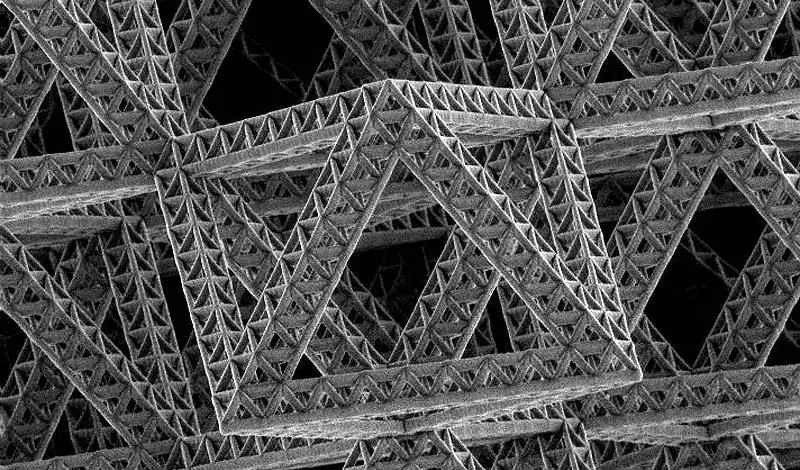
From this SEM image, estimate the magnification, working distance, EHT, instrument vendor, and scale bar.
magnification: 2.048 K X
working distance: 4.8 mm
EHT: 5 kV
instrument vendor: FEI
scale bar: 10000 nm